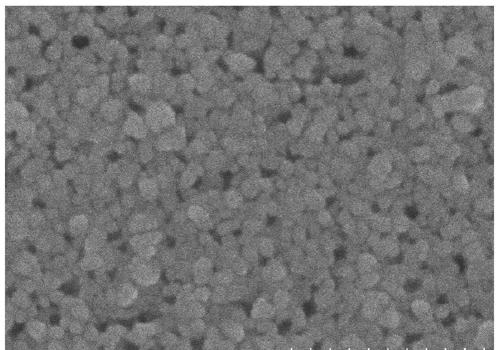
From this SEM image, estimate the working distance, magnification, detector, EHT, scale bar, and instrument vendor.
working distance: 10.2 mm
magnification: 50 K X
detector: SE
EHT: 20 kV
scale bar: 1000 nm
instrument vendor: Hitachi